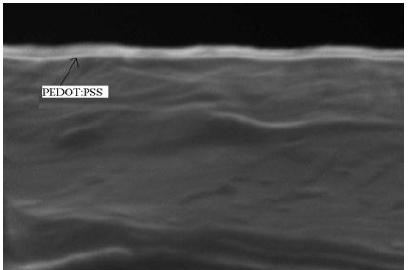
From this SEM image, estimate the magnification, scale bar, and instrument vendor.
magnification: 60 K X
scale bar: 200 nm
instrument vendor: JEOL